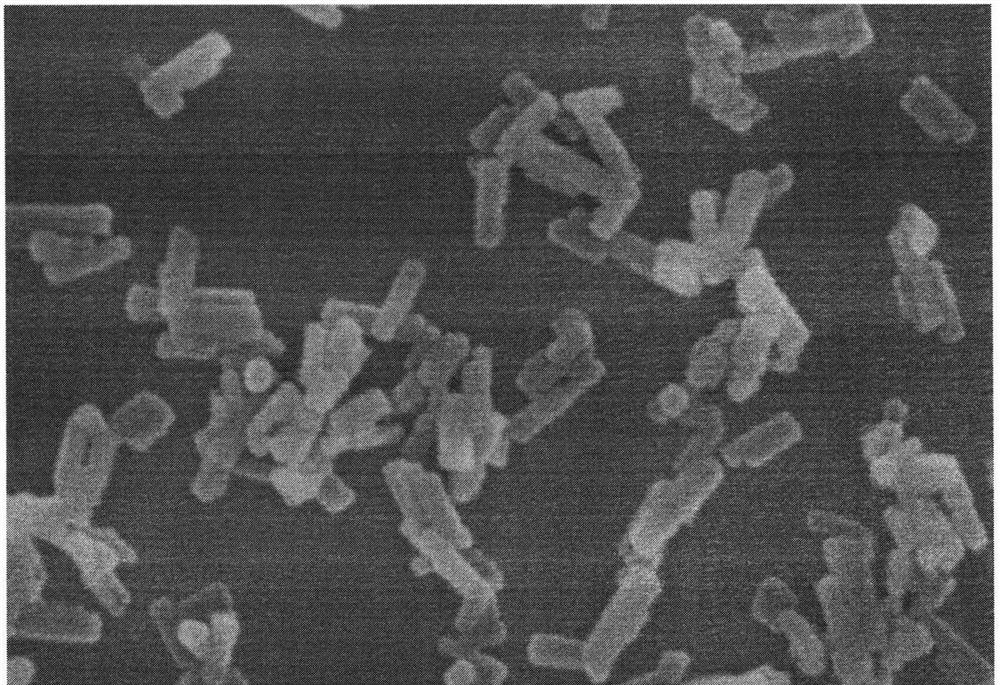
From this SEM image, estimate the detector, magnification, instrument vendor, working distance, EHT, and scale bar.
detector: InLens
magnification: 70 K X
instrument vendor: Zeiss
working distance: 9.3 mm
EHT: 10 kV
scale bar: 100 nm